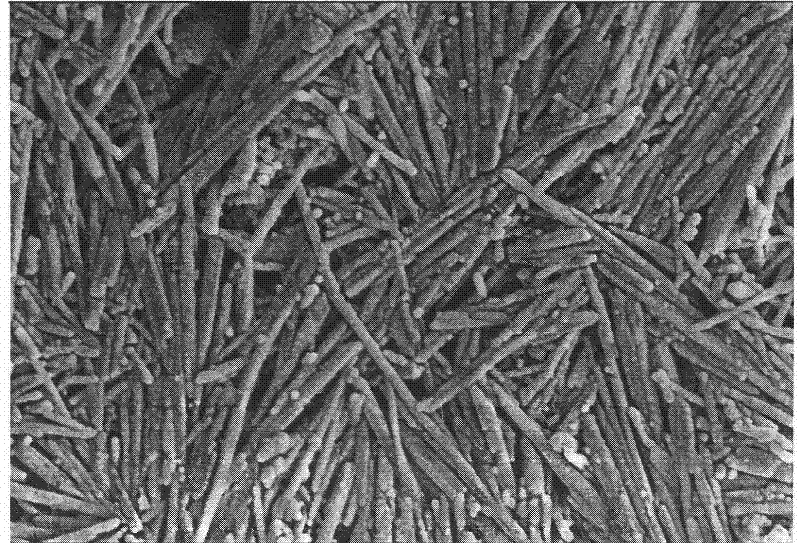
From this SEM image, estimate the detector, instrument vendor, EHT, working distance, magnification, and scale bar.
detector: SE(M)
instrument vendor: Hitachi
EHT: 5 kV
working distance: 8.4 mm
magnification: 30 K X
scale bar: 1000 nm